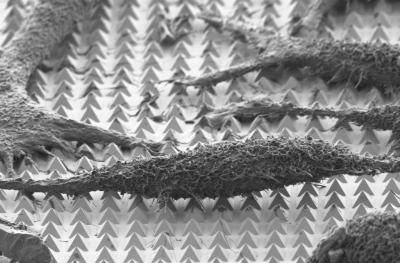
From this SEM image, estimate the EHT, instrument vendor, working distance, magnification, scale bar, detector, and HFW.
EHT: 1.5 kV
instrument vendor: Zeiss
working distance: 8.1 mm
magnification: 5.82 K X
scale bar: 2000 nm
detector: SE2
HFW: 51.51 µm